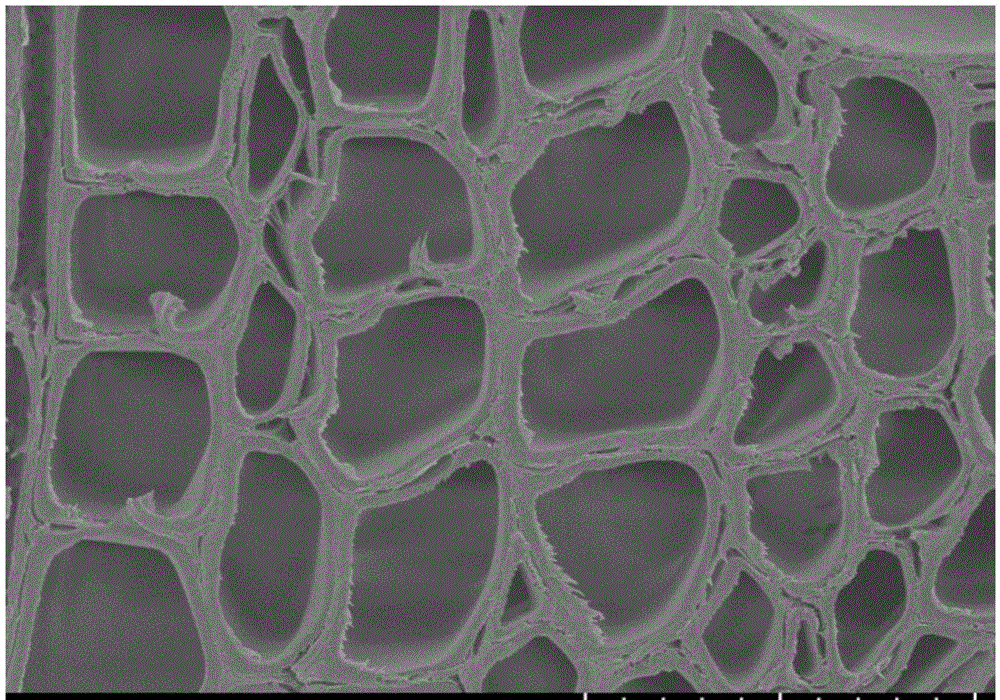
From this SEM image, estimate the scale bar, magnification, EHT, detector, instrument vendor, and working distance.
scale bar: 50000 nm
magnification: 0.8 K X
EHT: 5 kV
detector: SE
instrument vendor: Hitachi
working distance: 6.4 mm